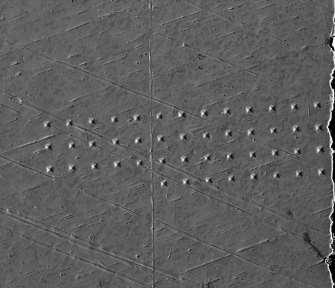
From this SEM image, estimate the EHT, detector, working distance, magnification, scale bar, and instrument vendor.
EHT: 20 kV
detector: ETD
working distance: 10 mm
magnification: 0.5 K X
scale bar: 100000 nm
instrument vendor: FEI